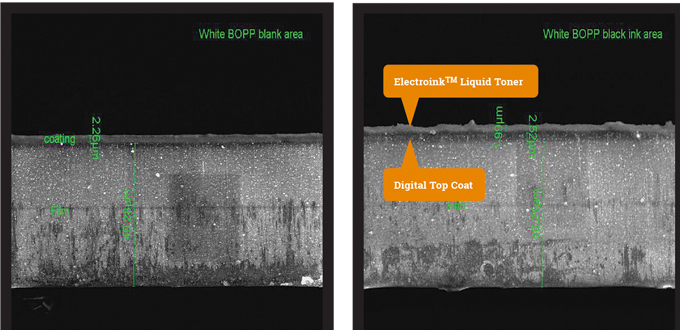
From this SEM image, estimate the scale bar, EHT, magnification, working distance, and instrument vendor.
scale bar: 50000 nm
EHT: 10 kV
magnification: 2 K X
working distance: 10.8 mm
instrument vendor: FEI